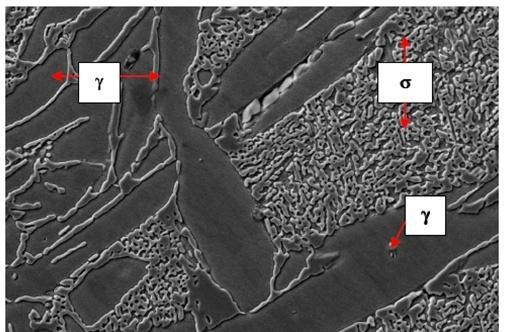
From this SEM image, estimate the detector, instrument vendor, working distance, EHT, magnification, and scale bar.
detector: SE1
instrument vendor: Zeiss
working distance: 25 mm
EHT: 20 kV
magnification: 2.5 K X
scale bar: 2000 nm